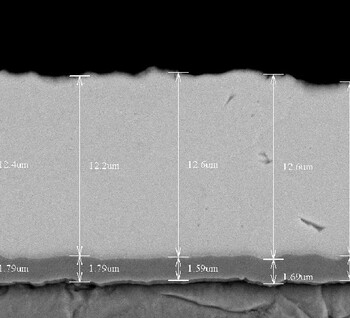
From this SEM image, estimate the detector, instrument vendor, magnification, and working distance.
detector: BSE3D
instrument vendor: Hitachi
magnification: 4 K X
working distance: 8.7 mm